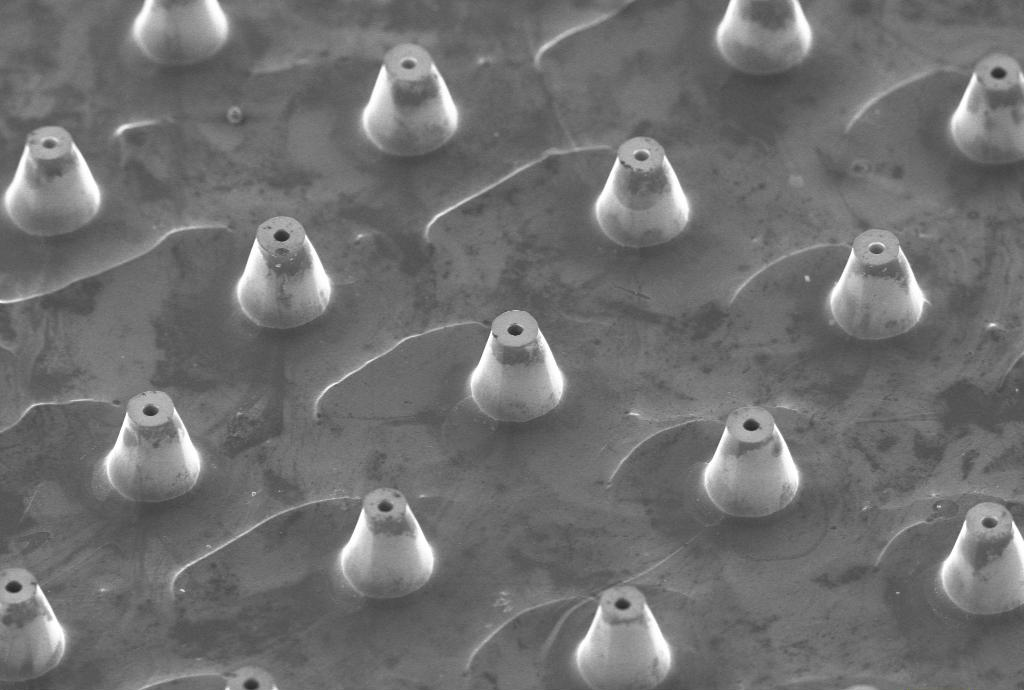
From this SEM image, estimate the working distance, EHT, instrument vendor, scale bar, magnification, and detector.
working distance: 9 mm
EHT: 10 kV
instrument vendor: Zeiss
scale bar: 100000 nm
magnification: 0.06 K X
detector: SE1